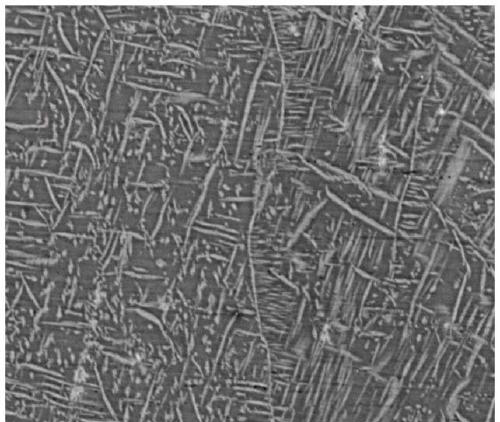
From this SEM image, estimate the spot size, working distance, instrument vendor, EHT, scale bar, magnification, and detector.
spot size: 6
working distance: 10 mm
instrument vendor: FEI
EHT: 20 kV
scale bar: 20000 nm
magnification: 5 K X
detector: DualBSD